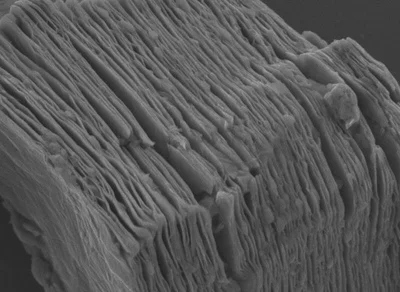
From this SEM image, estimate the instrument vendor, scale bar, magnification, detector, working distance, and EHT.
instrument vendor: JEOL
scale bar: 1000 nm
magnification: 20 K X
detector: LED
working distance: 10.3 mm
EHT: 4 kV